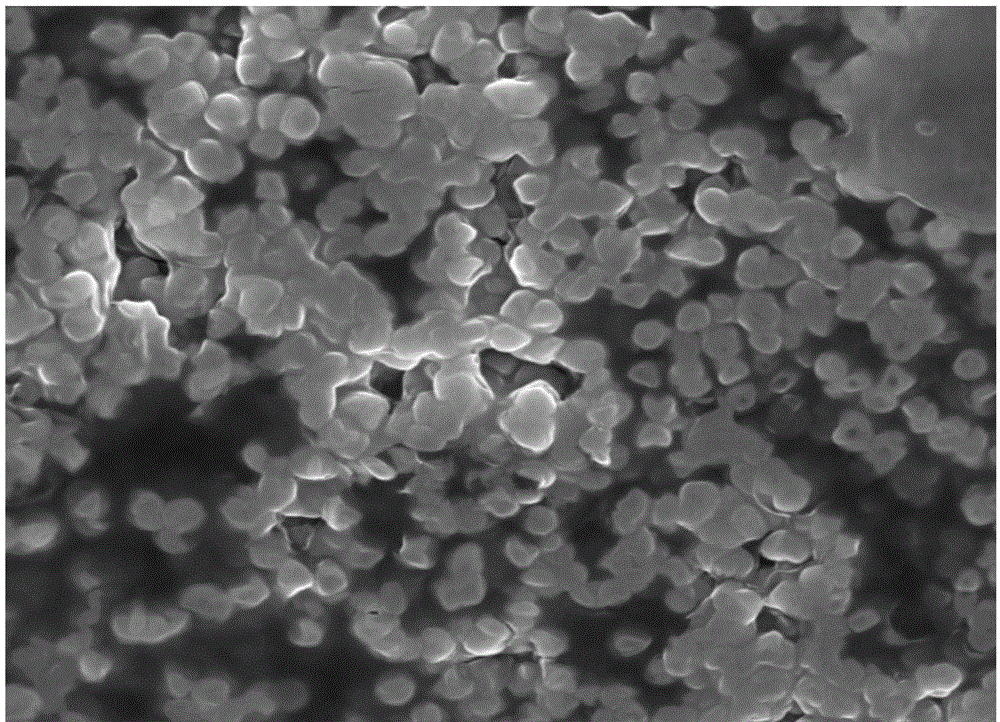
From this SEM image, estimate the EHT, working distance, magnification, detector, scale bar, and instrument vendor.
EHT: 10 kV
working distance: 5.7 mm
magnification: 30 K X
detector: TLD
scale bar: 3000 nm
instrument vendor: FEI